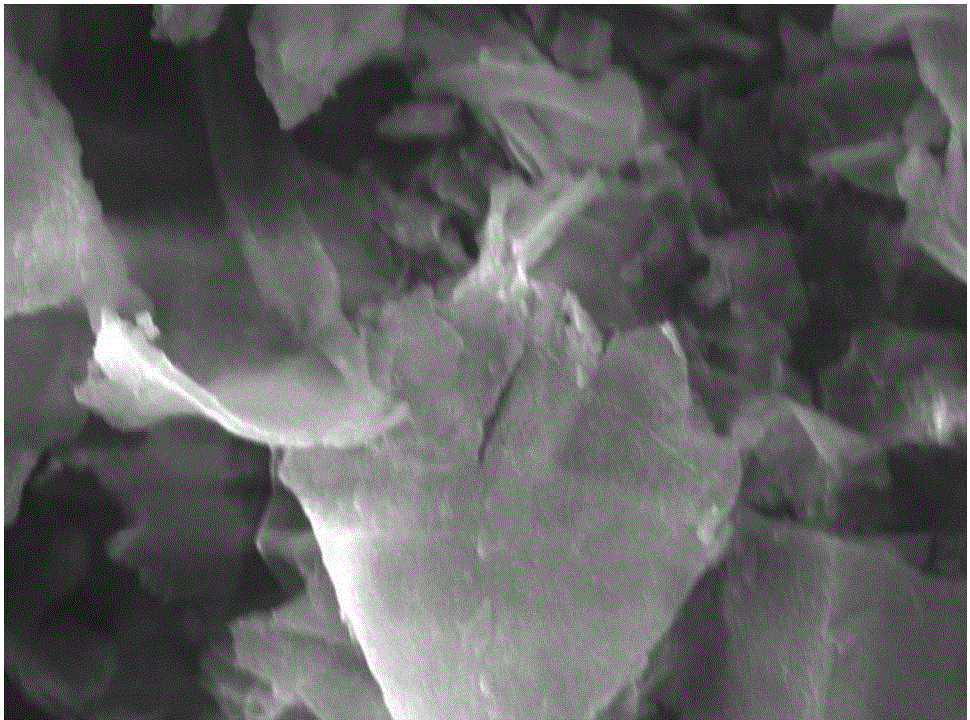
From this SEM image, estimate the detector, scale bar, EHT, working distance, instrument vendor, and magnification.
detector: SED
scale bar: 10000 nm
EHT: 20 kV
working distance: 10.6 mm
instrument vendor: JEOL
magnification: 2.5 K X